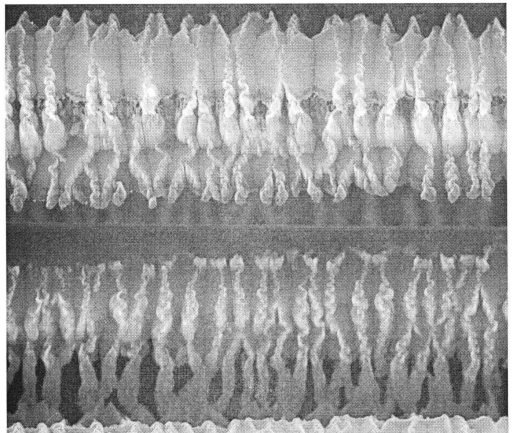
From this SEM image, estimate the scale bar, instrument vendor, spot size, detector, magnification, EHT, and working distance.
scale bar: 5000 nm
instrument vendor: FEI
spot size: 4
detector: ETD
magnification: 16 K X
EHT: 20 kV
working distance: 11.1 mm